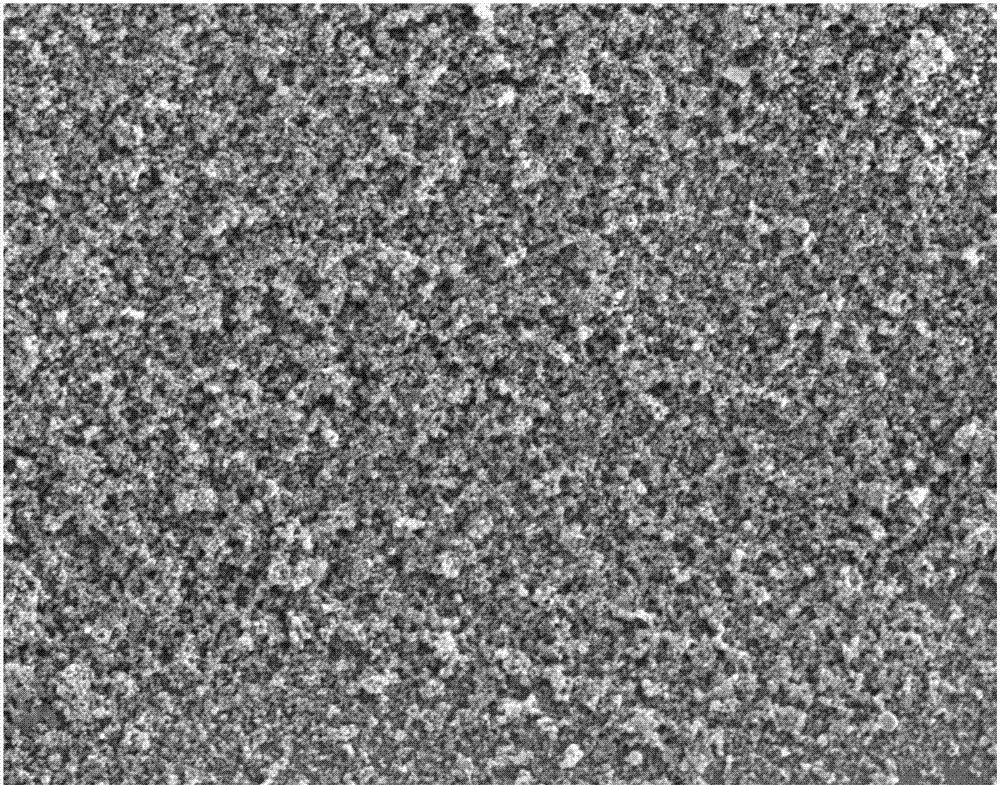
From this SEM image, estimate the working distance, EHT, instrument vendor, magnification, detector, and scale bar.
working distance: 5 mm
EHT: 5 kV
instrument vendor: FEI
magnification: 10 K X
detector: ETD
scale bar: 10000 nm